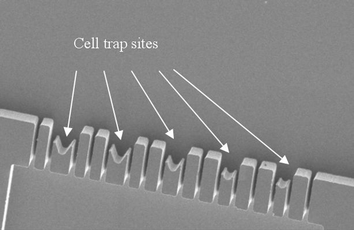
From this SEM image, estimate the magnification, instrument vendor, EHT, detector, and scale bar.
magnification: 0.6 K X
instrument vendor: JEOL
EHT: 2.5 kV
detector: SEI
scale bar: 20000 nm